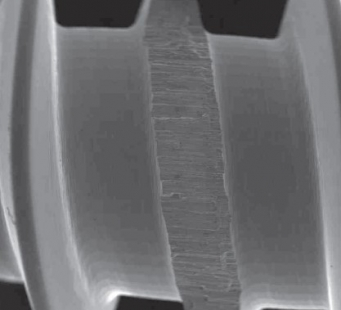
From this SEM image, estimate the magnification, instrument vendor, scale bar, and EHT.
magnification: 0.015 K X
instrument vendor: JEOL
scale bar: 1000000 nm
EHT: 20 kV